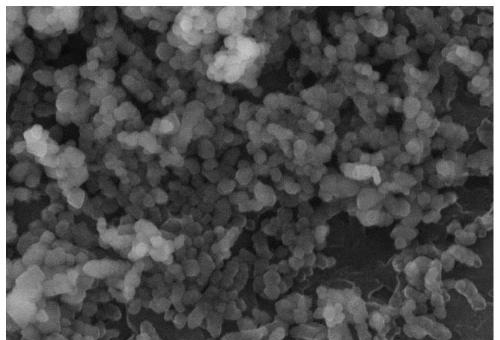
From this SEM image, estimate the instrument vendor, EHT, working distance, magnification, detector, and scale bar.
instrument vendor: Hitachi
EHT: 10 kV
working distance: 8.2 mm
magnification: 50 K X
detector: SE(TU)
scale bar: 1000 nm